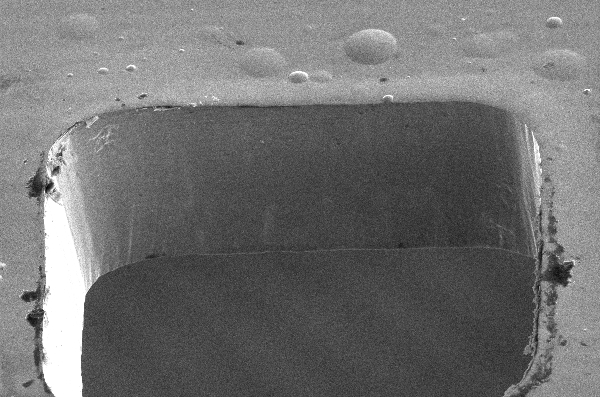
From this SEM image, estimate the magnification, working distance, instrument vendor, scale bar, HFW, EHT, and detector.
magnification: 0.35 K X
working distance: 8 mm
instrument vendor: FEI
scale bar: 100000 nm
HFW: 363 µm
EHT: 10 kV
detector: ETD